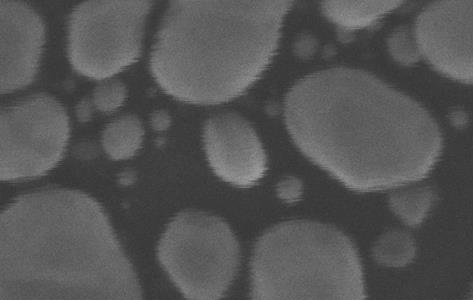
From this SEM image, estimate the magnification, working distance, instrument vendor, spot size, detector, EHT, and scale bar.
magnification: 646.363 K X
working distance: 6.1 mm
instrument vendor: FEI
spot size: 2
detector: TLD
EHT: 20 kV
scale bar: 50 nm